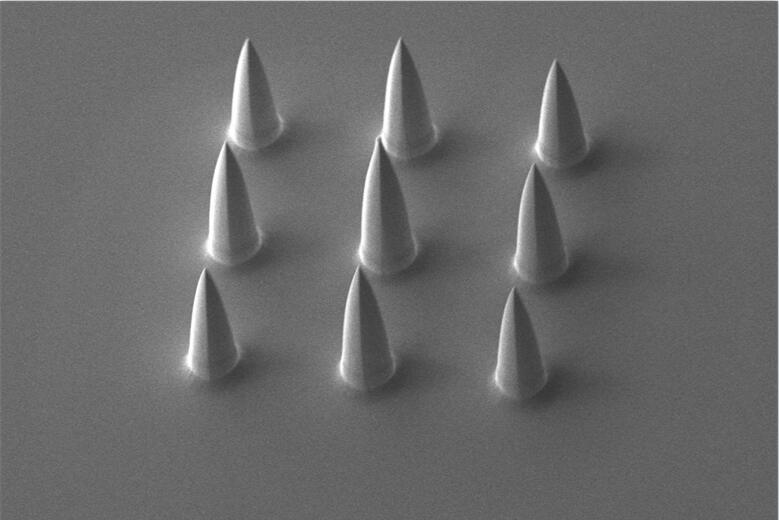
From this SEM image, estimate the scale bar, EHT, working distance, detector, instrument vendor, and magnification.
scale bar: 20000 nm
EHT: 10 kV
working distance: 23.5 mm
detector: SE1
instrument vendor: Zeiss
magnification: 0.243 K X